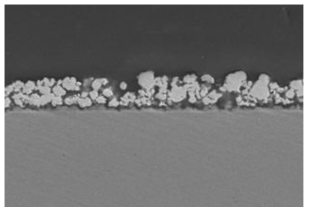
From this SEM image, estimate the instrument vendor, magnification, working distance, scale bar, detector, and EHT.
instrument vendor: Hitachi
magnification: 3 K X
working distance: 15 mm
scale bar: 10000 nm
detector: SE(M)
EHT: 15 kV